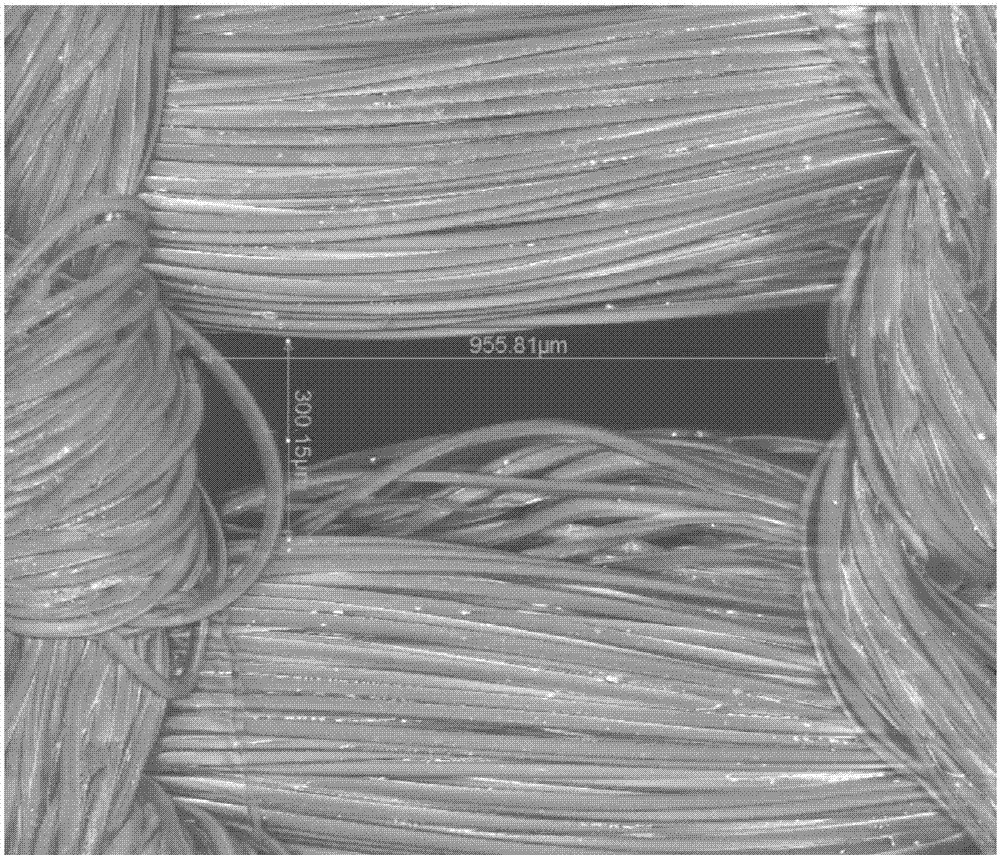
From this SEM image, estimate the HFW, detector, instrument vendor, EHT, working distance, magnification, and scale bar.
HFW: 1490 µm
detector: LFD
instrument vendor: FEI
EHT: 15 kV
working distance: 25.8 mm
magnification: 0.2 K X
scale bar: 500000 nm